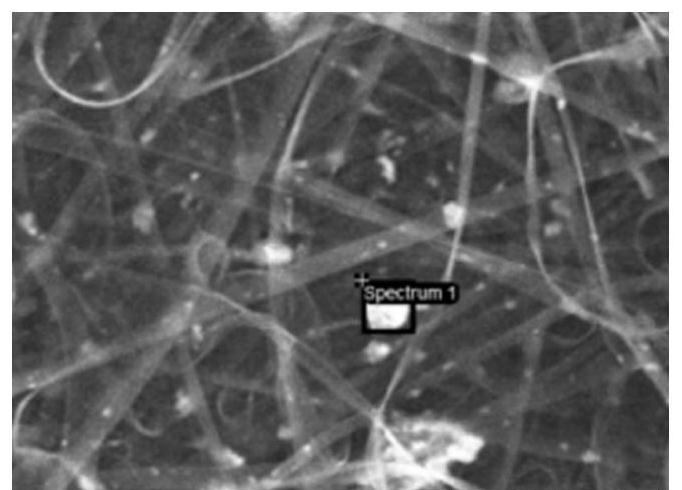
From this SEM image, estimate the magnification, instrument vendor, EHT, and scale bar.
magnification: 2 K X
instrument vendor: Hitachi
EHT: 5 kV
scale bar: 20000 nm